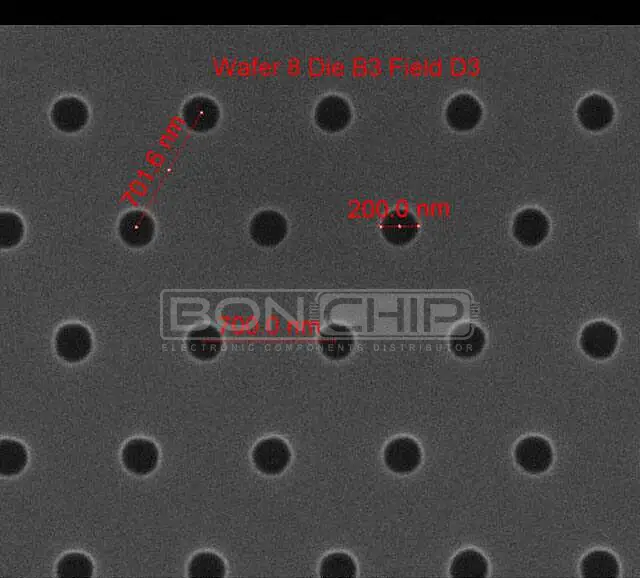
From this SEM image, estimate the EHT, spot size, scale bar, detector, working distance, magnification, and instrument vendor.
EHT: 10 kV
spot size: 1.5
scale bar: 1000 nm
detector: ETD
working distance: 10.4 mm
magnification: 75 K X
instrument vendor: FEI